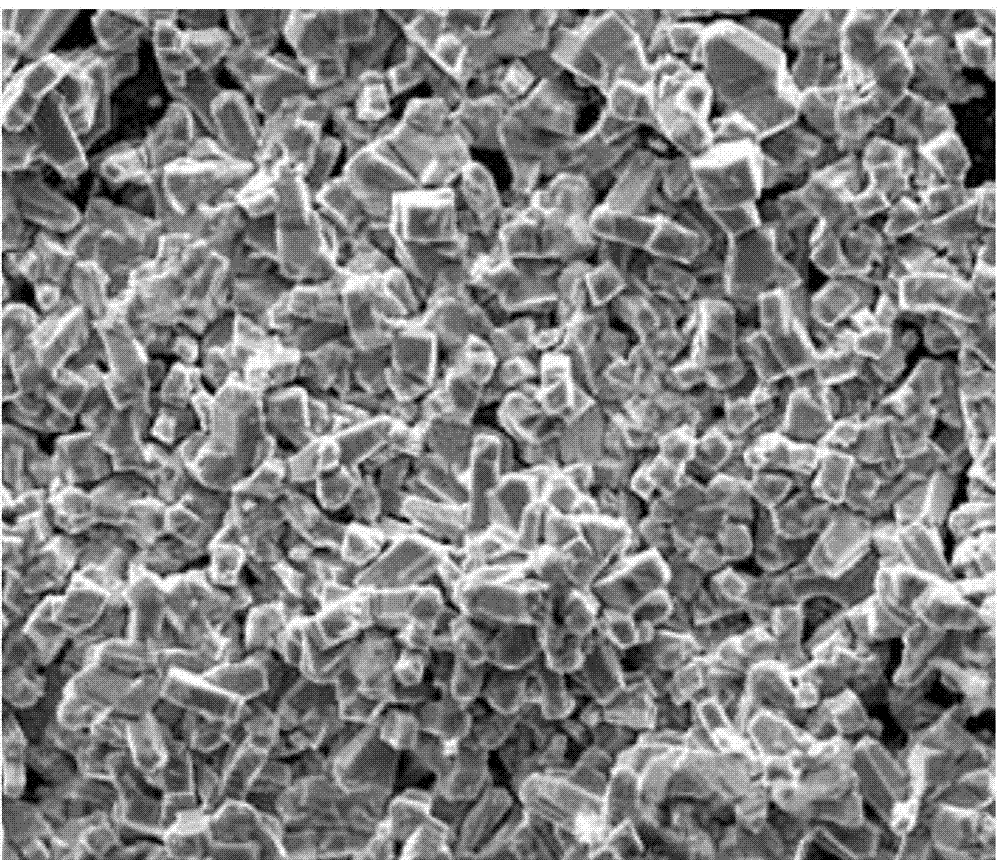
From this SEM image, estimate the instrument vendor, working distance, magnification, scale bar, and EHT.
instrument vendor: FEI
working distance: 16.1 mm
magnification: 2 K X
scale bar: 50000 nm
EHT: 20 kV